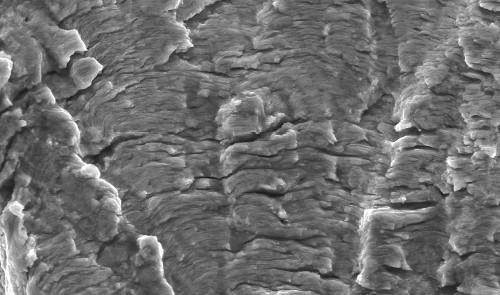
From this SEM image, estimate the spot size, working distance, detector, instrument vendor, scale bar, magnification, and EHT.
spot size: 3.2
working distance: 11.6 mm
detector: SE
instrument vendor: FEI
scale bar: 10000 nm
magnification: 2 K X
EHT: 20 kV